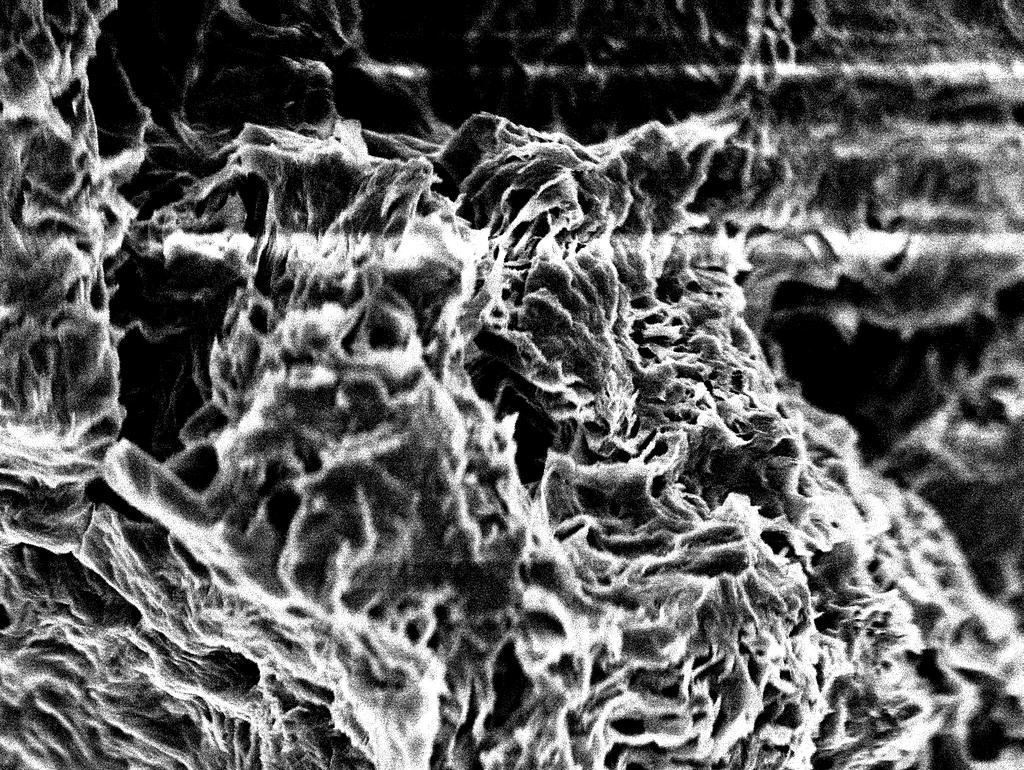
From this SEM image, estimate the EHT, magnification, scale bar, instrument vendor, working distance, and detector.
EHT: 10 kV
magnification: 0.65 K X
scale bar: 10000 nm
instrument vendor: JEOL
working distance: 9 mm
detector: SEI